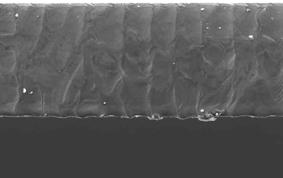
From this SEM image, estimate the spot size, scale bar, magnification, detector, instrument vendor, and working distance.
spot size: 4.5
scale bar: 300000 nm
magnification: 0.5 K X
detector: SE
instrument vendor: FEI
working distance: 10.5 mm